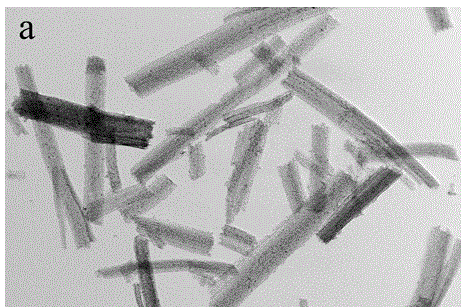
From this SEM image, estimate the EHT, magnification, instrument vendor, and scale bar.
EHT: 200 kV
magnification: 15 K X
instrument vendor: JEOL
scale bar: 500 nm